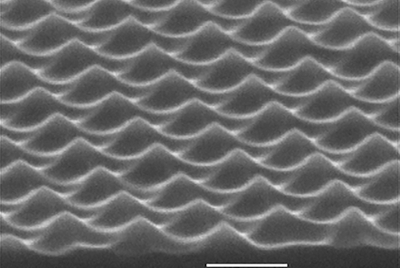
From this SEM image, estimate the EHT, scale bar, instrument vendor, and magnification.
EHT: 15 kV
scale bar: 500 nm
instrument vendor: JEOL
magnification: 50 K X